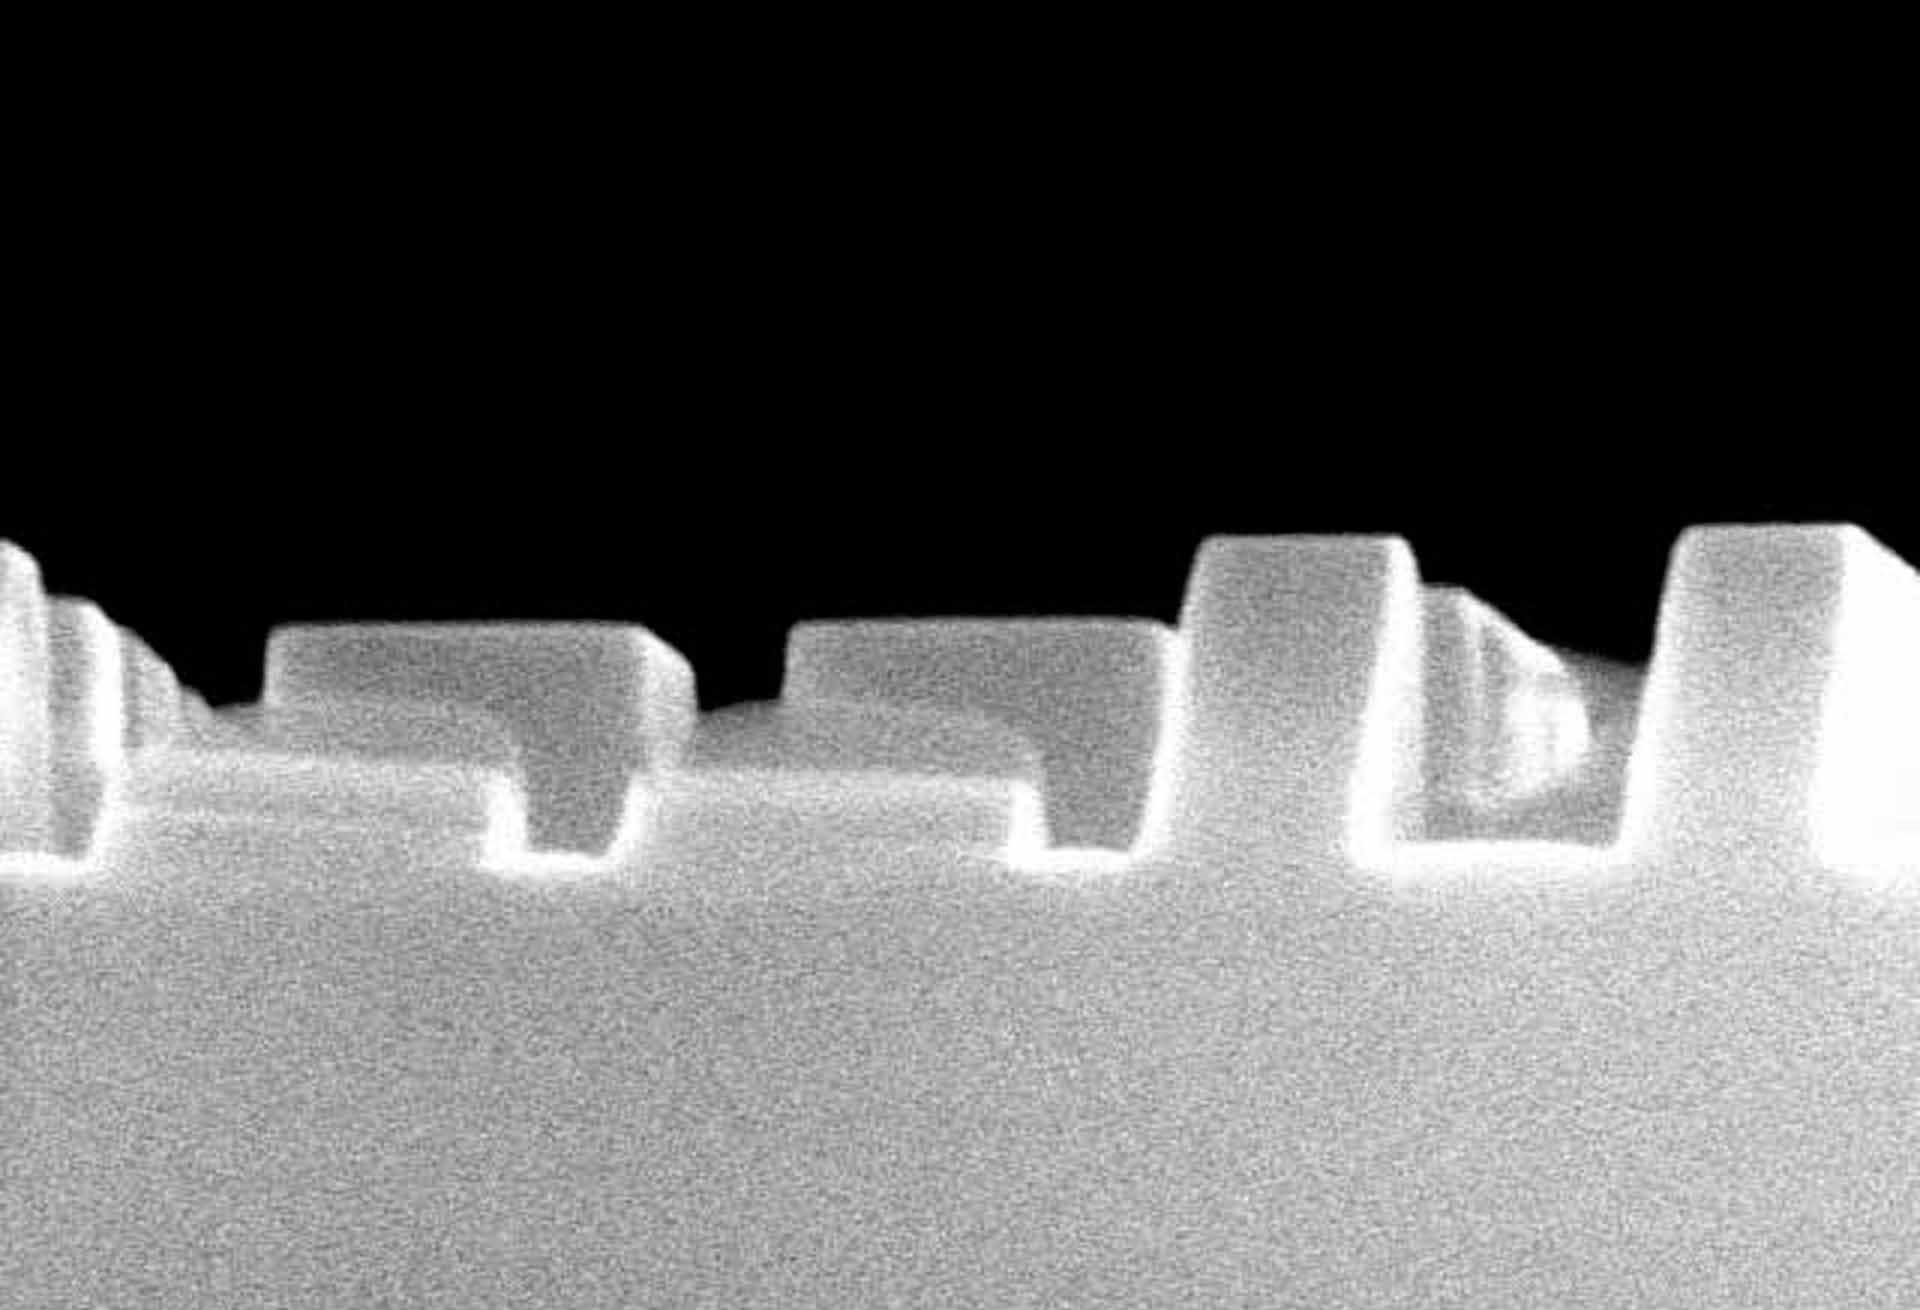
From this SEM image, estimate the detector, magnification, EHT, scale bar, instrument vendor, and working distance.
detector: SE(M)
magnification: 80.1 K X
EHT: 15 kV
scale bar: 500 nm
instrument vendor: Hitachi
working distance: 15.7 mm